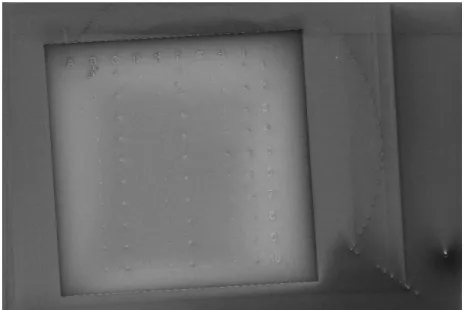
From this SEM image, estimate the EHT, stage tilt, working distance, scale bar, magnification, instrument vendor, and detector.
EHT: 5 kV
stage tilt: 0°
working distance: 4.9 mm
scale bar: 100000 nm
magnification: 0.179 K X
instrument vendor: Zeiss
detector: InLens